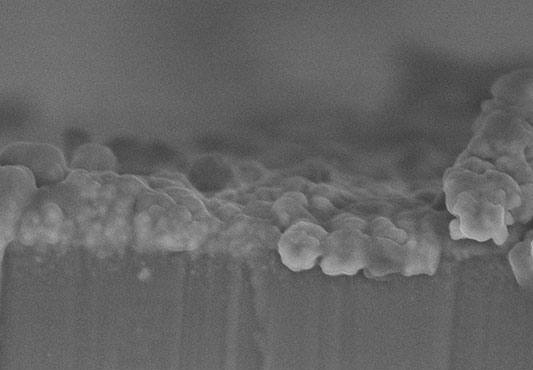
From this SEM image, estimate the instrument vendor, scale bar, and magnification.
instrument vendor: Hitachi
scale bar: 1000 nm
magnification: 50 K X